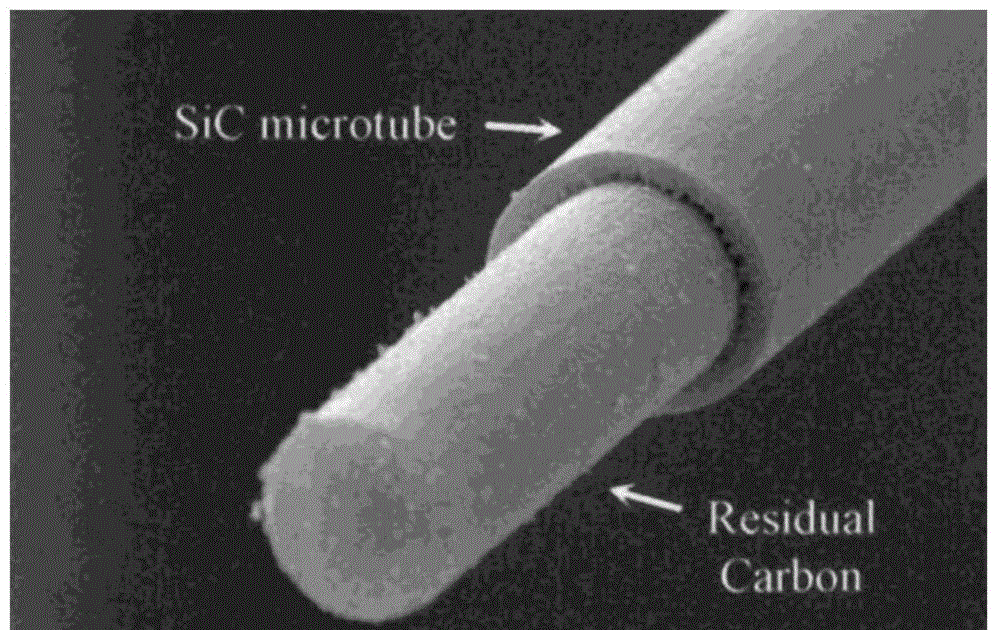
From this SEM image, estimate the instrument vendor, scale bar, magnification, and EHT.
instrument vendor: JEOL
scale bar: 5000 nm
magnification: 5 K X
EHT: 20 kV